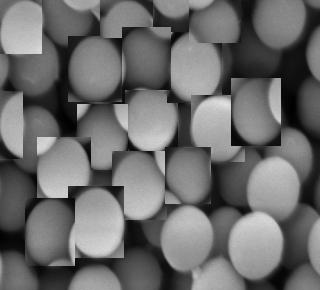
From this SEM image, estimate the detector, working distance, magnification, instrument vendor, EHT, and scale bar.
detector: LED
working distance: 7.8 mm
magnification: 50 K X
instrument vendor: JEOL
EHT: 5 kV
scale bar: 100 nm